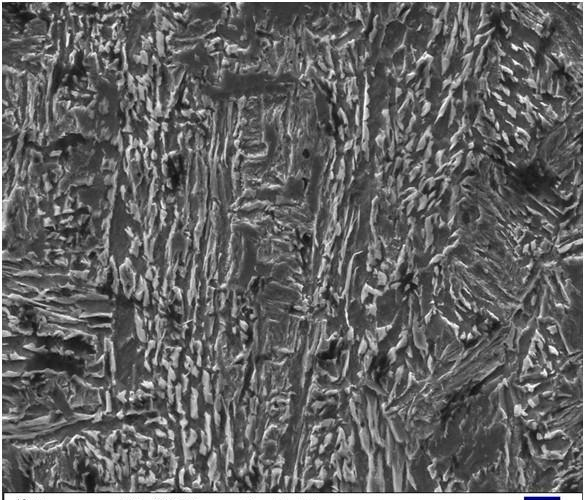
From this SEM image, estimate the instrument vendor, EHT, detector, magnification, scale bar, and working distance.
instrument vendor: Zeiss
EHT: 20 kV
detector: SE1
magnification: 1 K X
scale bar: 10000 nm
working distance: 12.5 mm